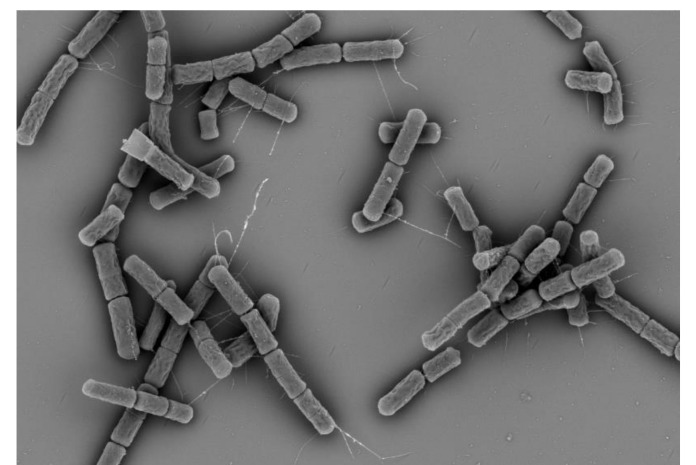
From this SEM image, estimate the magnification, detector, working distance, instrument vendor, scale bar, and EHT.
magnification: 4.5 K X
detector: BSE-ALL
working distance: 6 mm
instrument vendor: Hitachi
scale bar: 10000 nm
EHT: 5 kV